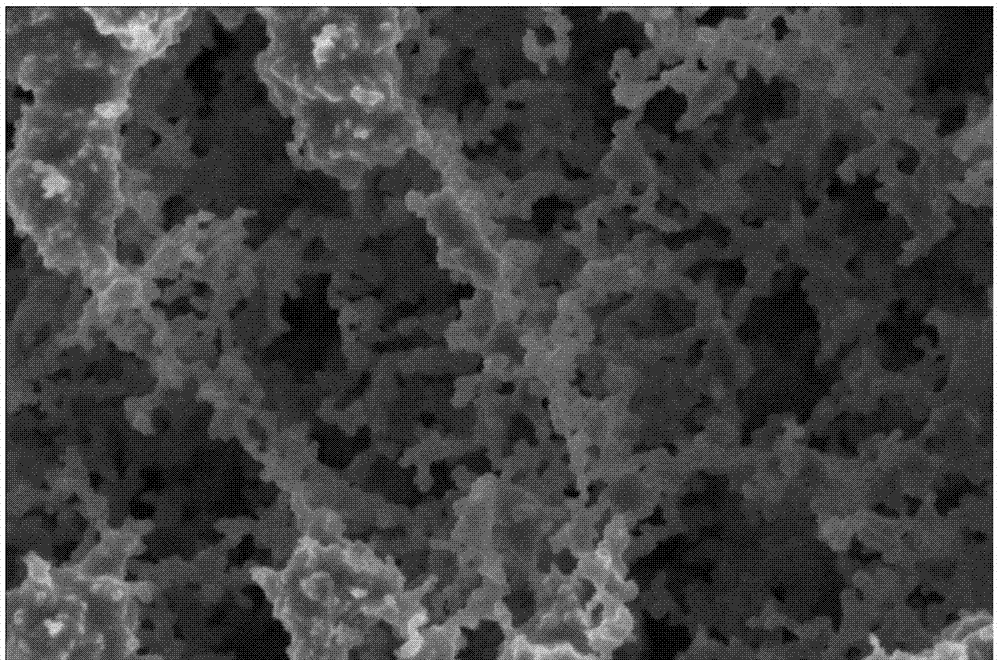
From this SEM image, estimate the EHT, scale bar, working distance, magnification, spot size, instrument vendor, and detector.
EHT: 3 kV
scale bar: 2000 nm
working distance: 5 mm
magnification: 80 K X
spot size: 3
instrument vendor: FEI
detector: TLD